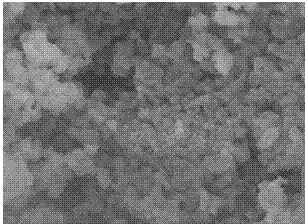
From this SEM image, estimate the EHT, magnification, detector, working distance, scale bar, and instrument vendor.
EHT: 7 kV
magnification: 50 K X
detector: SE(M)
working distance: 9 mm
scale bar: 1000 nm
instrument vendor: Hitachi